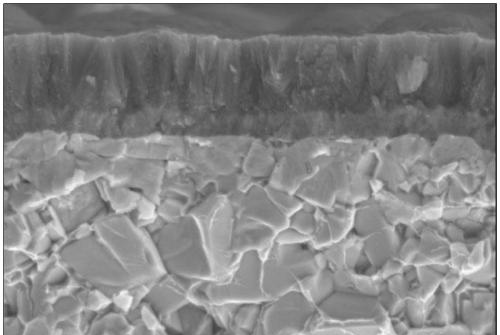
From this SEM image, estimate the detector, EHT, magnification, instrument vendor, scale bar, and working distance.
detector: SE2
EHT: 10 kV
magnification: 10 K X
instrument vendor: Zeiss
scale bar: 1000 nm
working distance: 20.7 mm